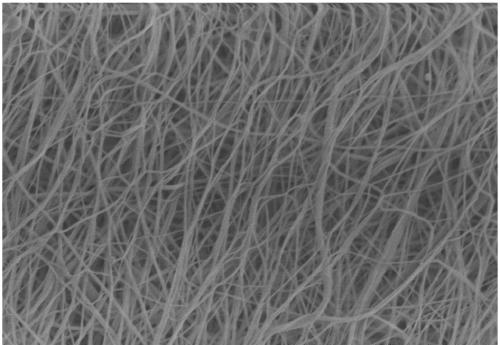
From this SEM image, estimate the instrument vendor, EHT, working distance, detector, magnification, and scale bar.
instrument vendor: Hitachi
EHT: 3 kV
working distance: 13 mm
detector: SE(M)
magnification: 10 K X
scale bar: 5000 nm